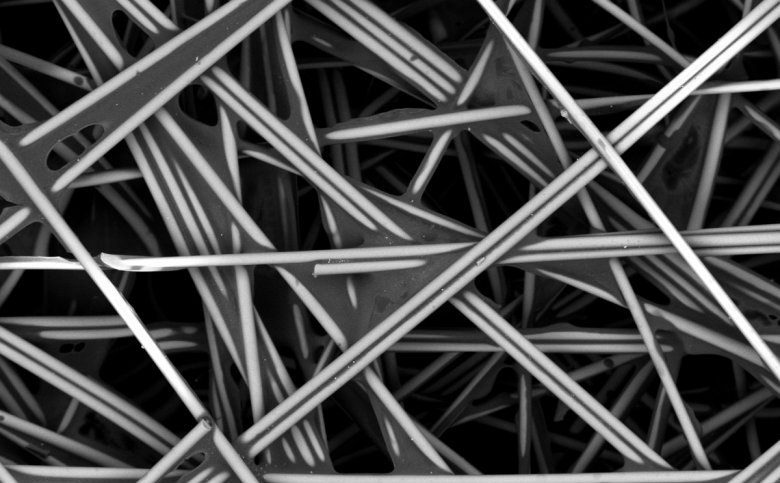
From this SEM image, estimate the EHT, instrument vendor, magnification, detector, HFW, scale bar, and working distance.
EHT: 10 kV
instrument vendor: other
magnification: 0.2 K X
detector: BSD Full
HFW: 635 µm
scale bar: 200000 nm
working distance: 7.092 mm